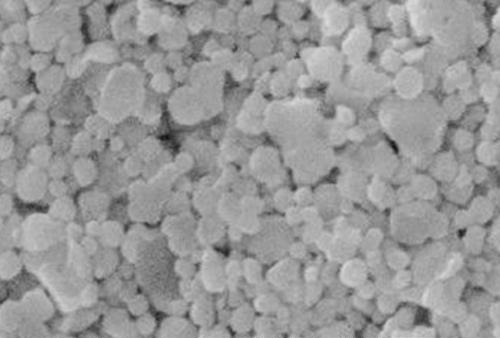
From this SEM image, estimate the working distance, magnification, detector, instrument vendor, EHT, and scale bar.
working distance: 9.7 mm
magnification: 10 K X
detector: ETD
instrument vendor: FEI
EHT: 20 kV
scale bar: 100 nm